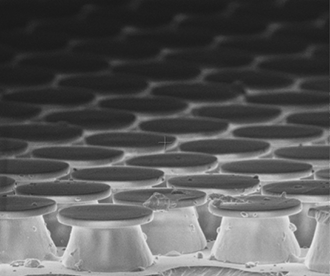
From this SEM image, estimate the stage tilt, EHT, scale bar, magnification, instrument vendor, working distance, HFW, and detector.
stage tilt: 10°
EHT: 2 kV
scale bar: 10000 nm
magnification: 5 K X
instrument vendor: FEI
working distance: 5.8 mm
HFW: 59.7 µm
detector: TLD